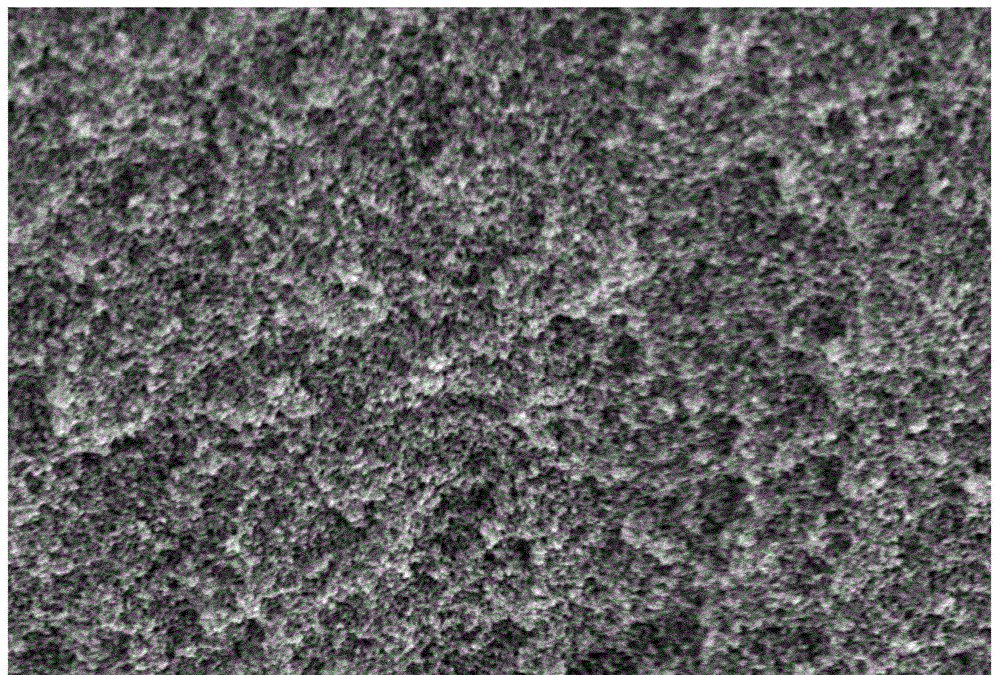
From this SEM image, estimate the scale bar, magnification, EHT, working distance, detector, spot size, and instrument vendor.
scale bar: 1000 nm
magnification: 10 K X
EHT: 15 kV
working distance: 11 mm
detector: SEI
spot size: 30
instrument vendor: JEOL